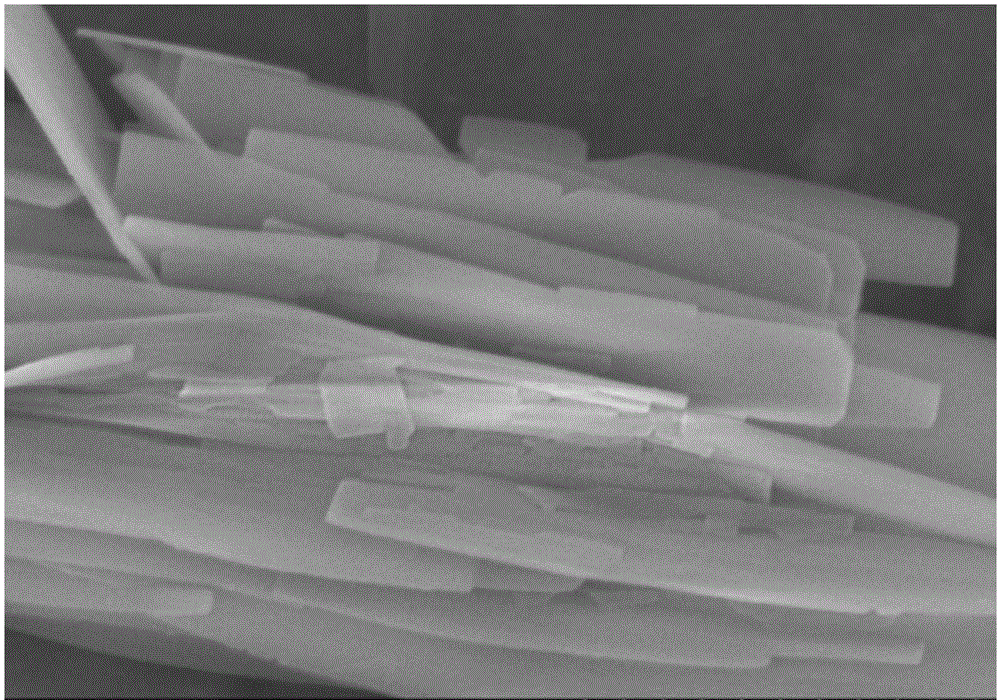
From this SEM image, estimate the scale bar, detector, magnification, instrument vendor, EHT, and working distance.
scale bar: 1000 nm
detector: SE(M)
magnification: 50 K X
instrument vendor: Hitachi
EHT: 5 kV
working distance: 5.4 mm